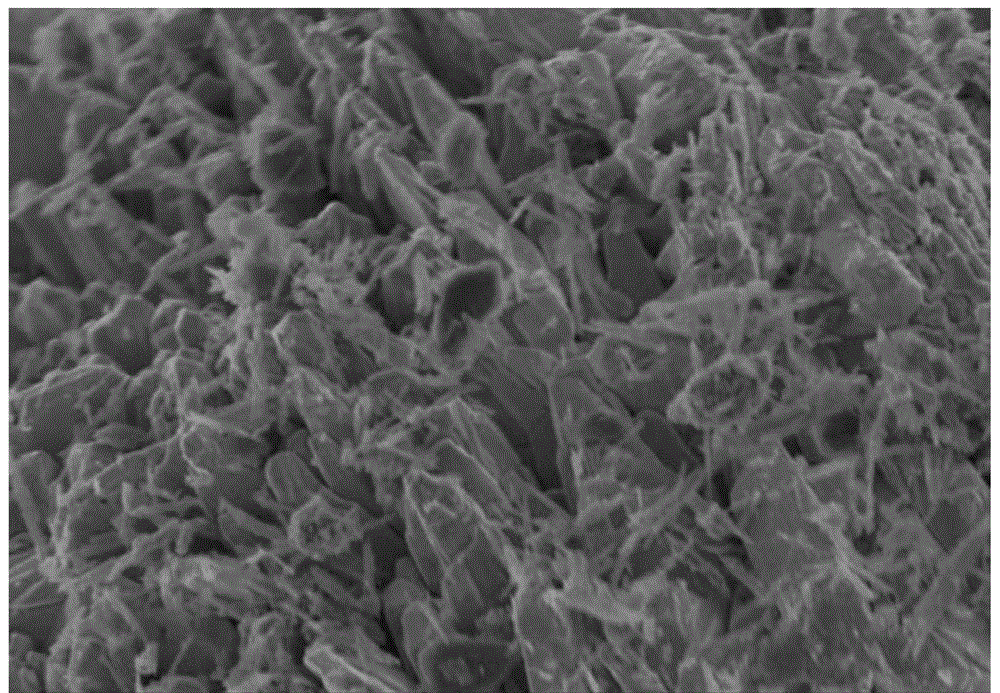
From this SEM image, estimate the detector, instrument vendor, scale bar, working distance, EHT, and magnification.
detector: SE(M)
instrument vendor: Hitachi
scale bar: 2000 nm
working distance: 5.6 mm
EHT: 2 kV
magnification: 20 K X